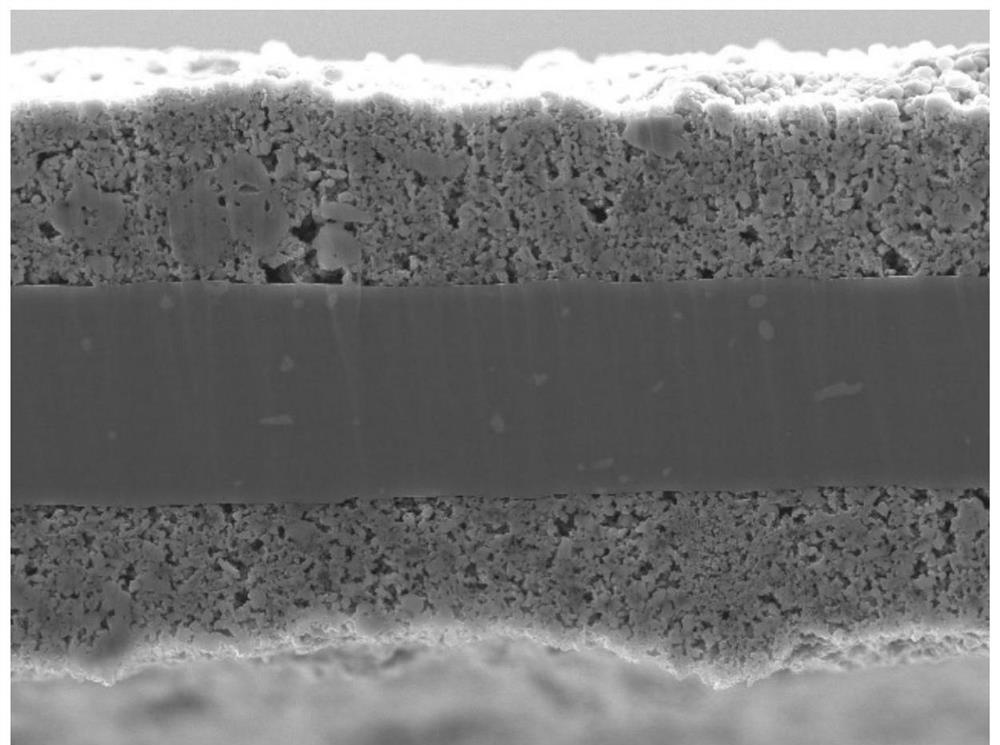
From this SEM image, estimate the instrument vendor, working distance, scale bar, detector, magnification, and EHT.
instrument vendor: JEOL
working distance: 10 mm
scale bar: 1000 nm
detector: LED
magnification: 3 K X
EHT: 10 kV